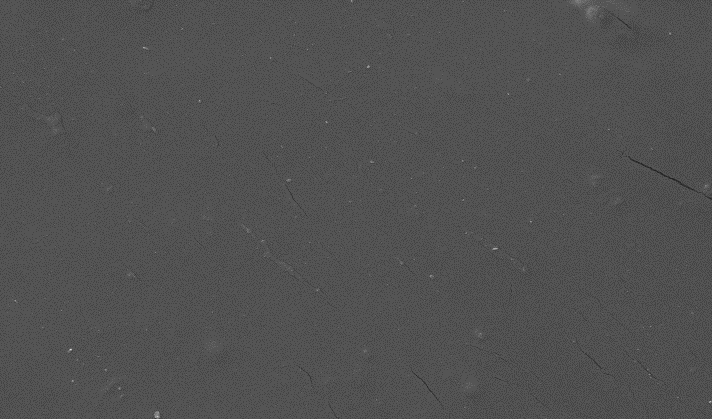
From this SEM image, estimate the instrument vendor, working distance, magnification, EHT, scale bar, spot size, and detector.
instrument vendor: FEI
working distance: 10.7 mm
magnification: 0.5 K X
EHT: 10 kV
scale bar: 50000 nm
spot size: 3.5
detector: SE